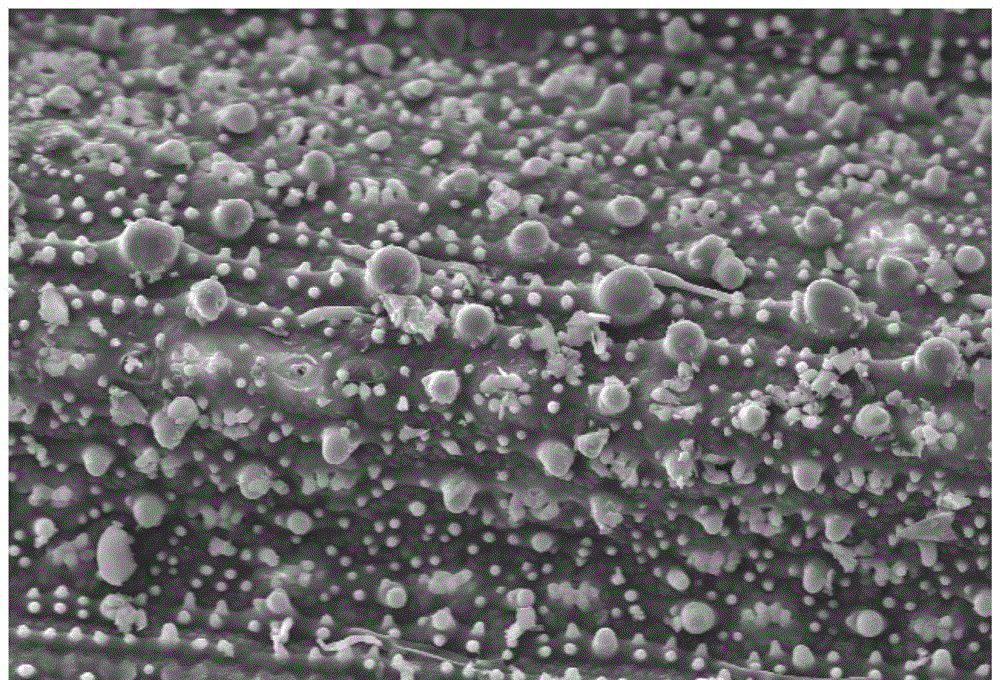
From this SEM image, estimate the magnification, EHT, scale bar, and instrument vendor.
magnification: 0.5 K X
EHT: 20 kV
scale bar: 50000 nm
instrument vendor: JEOL